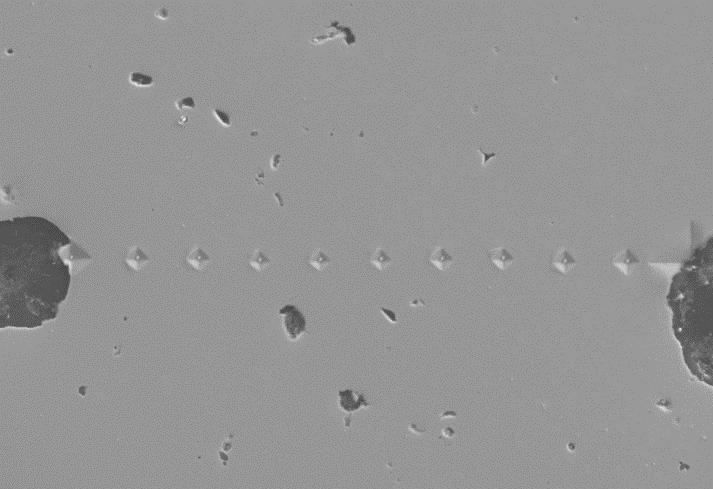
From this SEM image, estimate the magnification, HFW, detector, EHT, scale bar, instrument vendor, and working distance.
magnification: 0.5 K X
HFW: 228.7 µm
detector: SE2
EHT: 20 kV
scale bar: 10000 nm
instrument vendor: Zeiss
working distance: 10.5 mm